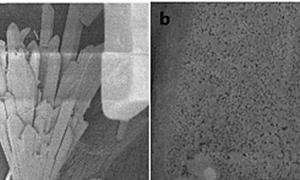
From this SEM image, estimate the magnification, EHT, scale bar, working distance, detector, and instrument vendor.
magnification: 0.6 K X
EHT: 8 kV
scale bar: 10000 nm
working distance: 8.2 mm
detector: SEI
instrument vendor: JEOL